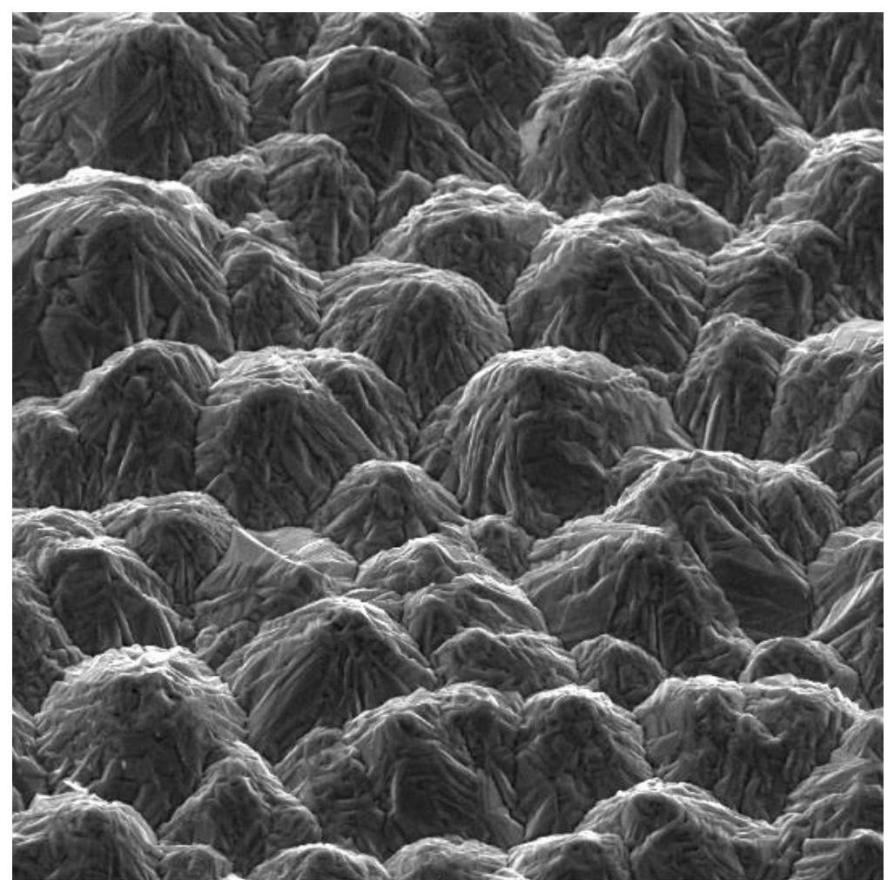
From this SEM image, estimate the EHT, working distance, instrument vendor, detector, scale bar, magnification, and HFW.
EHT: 20 kV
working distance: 21.17 mm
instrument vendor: Tescan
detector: SE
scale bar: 20000 nm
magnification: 2 K X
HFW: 104 µm